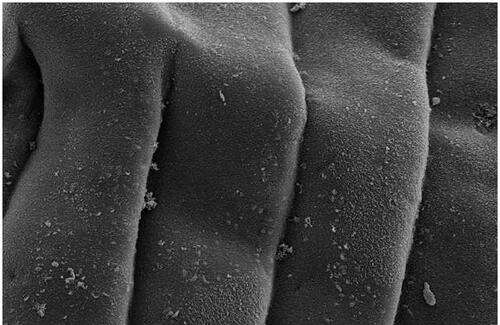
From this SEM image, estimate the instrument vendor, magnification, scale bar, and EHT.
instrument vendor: JEOL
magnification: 0.75 K X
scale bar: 20000 nm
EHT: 20 kV